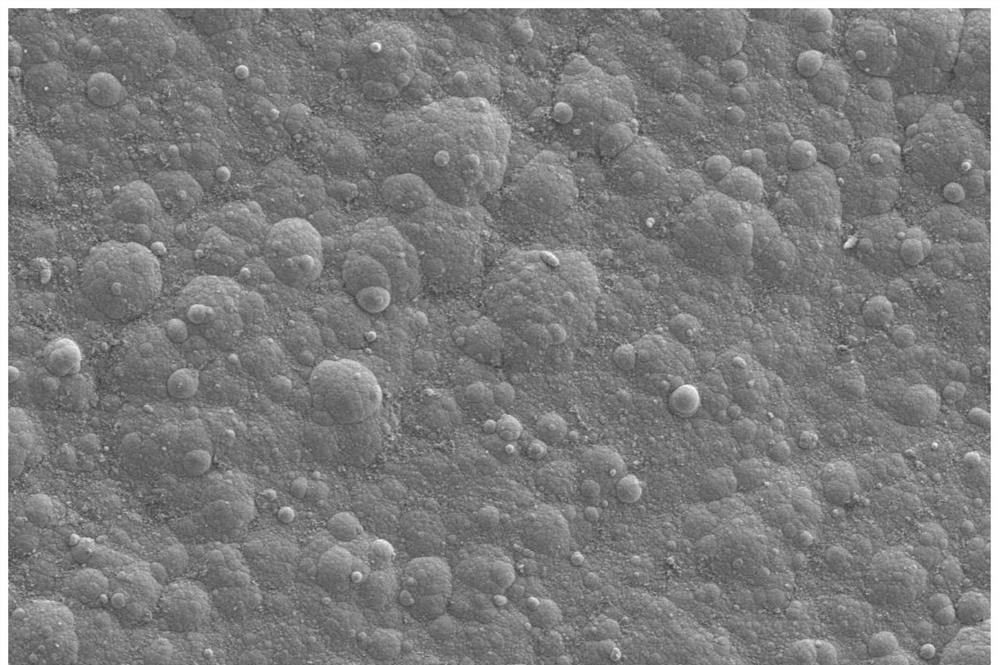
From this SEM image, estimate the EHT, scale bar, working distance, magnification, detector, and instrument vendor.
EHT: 5 kV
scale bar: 2000 nm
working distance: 6.2 mm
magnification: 2 K X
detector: SE2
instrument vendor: Zeiss